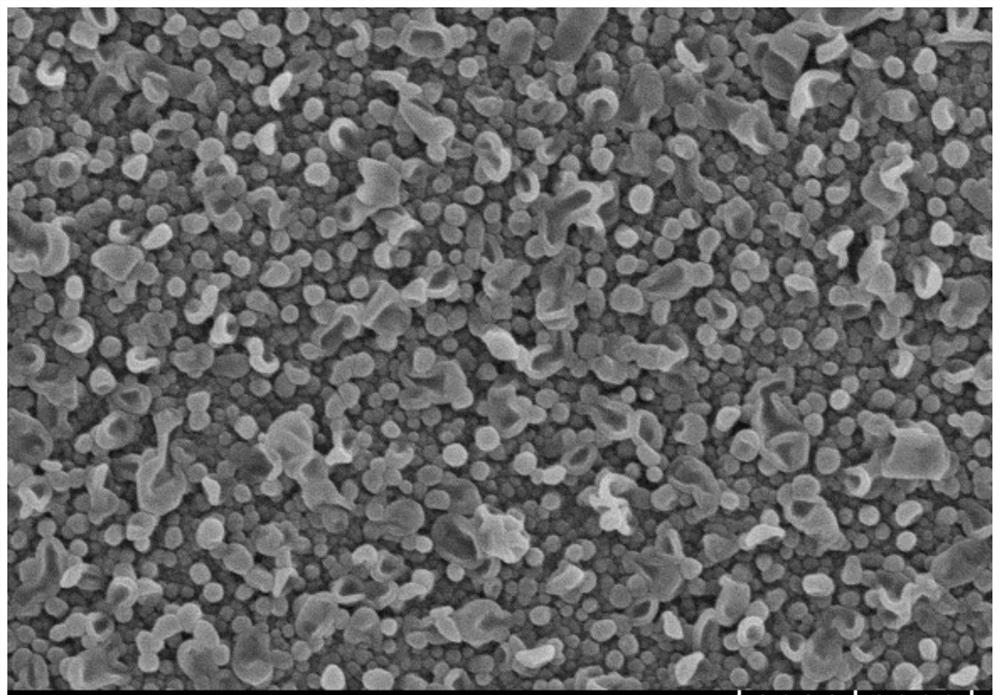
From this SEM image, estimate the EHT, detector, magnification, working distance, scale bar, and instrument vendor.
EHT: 3 kV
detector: SE(UL)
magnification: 30 K X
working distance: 9.2 mm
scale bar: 1000 nm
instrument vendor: Hitachi